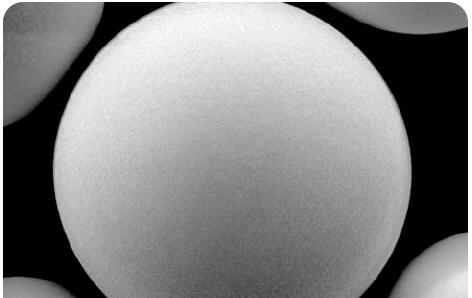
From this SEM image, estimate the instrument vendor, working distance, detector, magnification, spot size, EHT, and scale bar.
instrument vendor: FEI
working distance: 11.3 mm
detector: SE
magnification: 4.5 K X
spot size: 3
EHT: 5 kV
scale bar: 5000 nm